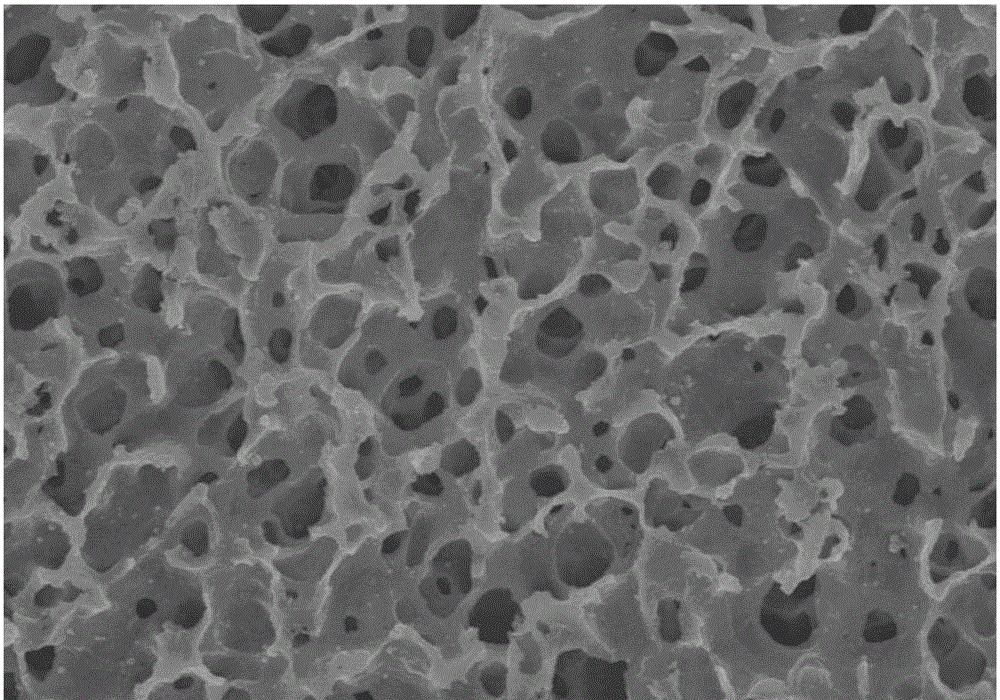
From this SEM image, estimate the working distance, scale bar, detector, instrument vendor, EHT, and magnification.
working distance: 6.4 mm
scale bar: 20000 nm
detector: SE(M)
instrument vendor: Hitachi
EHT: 15 kV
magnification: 2 K X